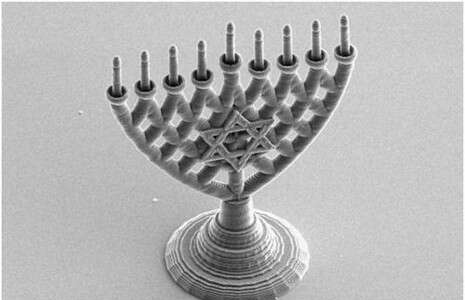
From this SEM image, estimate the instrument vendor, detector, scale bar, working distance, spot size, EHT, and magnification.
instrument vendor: FEI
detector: SE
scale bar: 20000 nm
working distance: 5.1 mm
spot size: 3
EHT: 5 kV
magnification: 1 K X